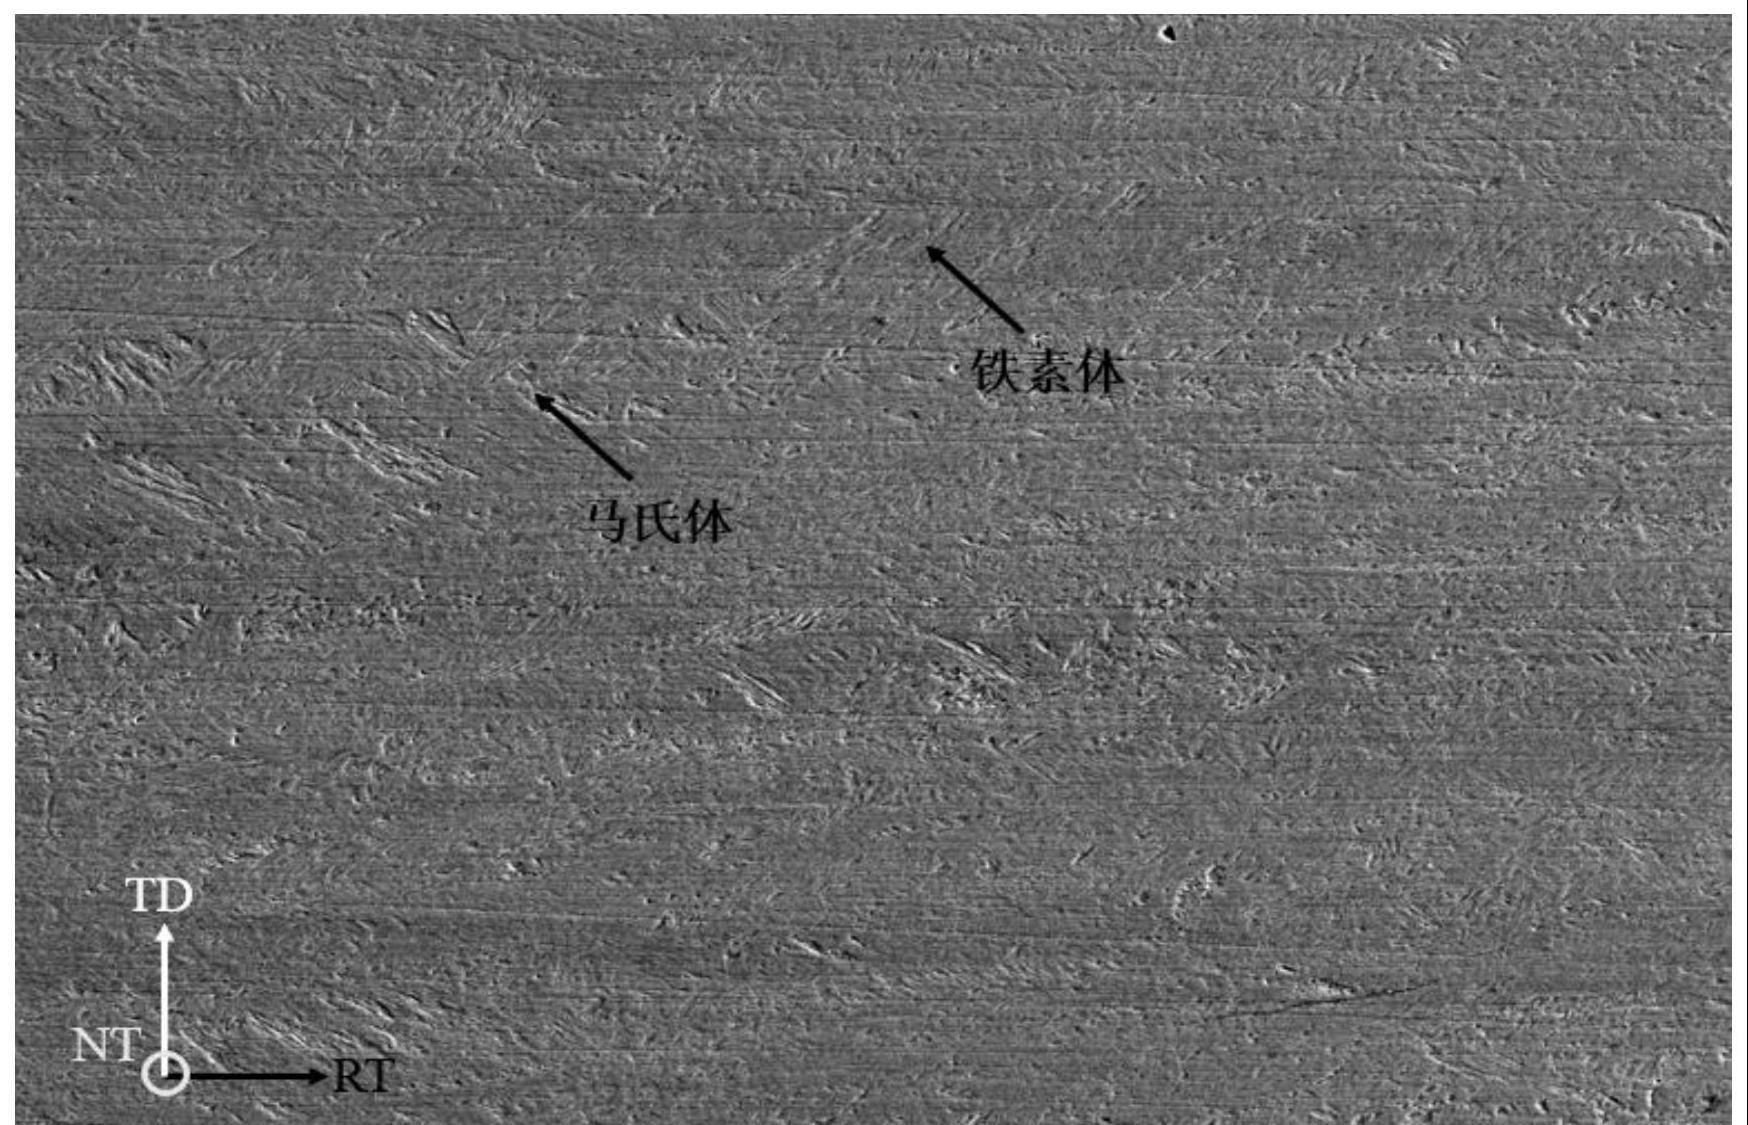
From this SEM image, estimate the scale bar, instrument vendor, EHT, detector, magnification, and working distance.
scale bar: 100000 nm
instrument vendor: Hitachi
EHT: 20 kV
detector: SE(L)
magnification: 0.5 K X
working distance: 18.4 mm